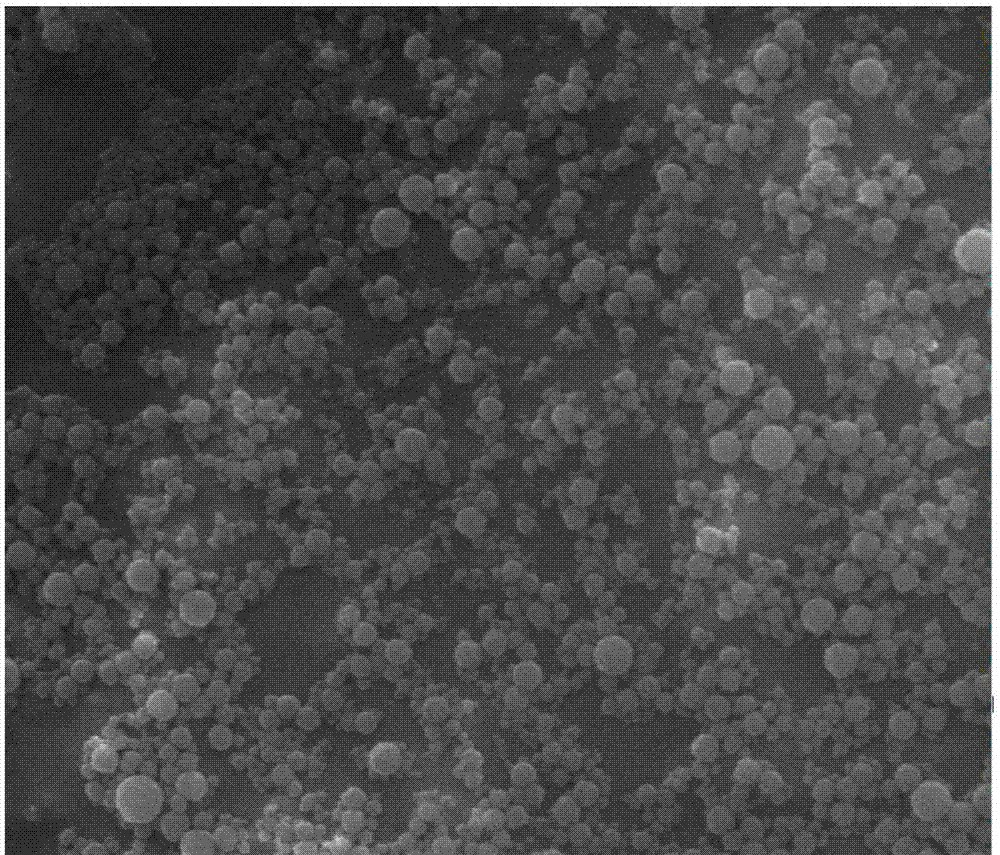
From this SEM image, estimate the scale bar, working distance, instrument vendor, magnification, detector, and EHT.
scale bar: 1000 nm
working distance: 10.5 mm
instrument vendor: FEI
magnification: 80 K X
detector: SE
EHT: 20 kV